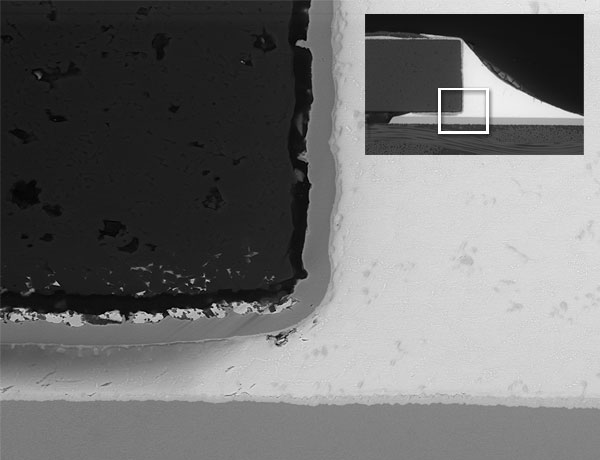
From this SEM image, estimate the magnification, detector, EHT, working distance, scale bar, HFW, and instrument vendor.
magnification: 2 K X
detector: BSED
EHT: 20 kV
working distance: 10.2 mm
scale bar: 40000 nm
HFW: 149 µm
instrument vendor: FEI